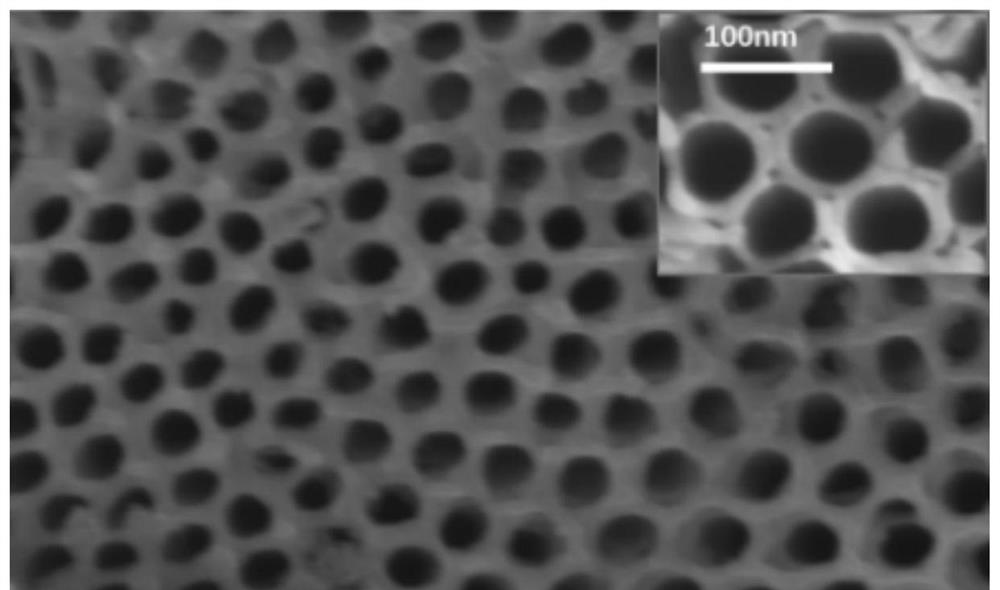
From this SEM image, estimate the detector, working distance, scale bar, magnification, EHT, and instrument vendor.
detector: SEI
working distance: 8.3 mm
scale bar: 100 nm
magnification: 95 K X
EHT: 5 kV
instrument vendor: JEOL